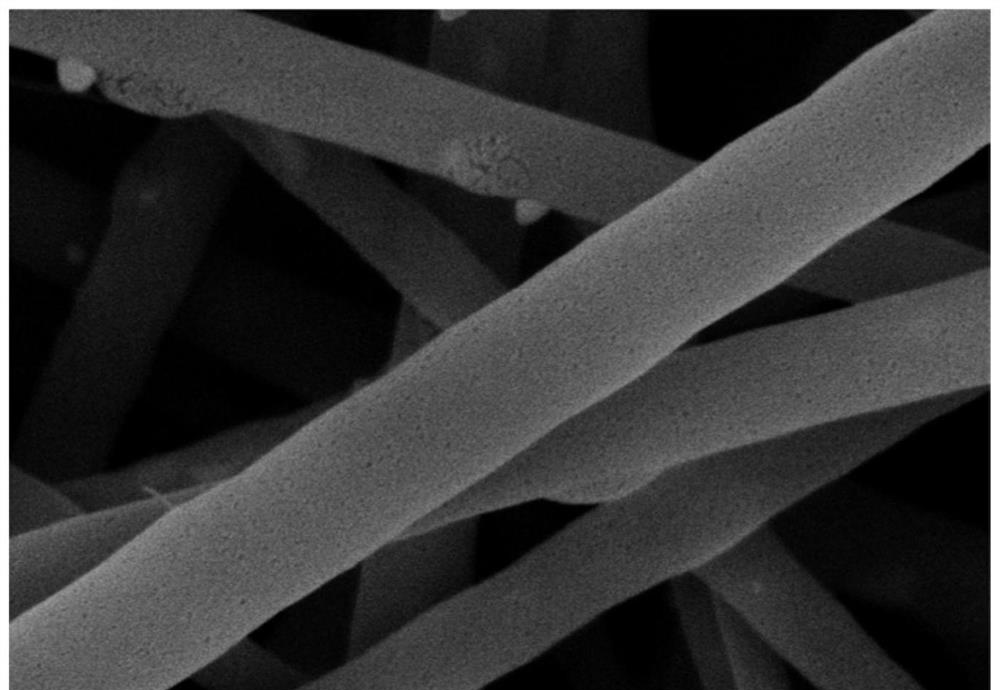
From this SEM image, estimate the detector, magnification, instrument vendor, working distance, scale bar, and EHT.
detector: SE(L)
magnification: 50 K X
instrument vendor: Hitachi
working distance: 9.3 mm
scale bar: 1000 nm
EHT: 5 kV